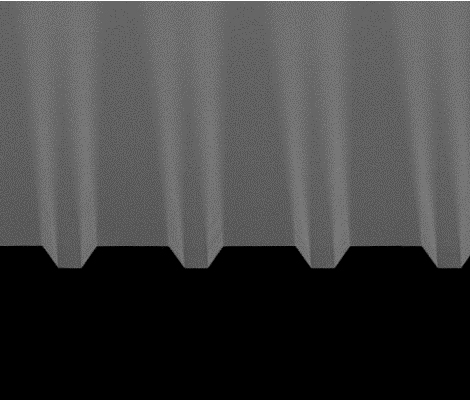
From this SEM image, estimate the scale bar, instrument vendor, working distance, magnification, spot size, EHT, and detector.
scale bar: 10000 nm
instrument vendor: FEI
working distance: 4.5 mm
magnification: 2.498 K X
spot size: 2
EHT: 10 kV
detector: SE